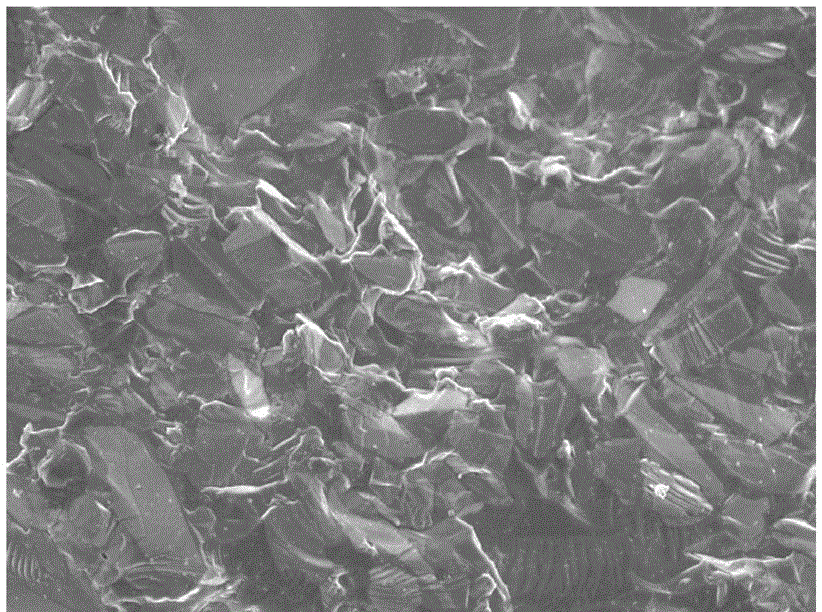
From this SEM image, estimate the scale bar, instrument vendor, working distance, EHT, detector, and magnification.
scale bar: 1000 nm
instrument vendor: JEOL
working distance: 7.7 mm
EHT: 5 kV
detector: SEI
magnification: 3 K X